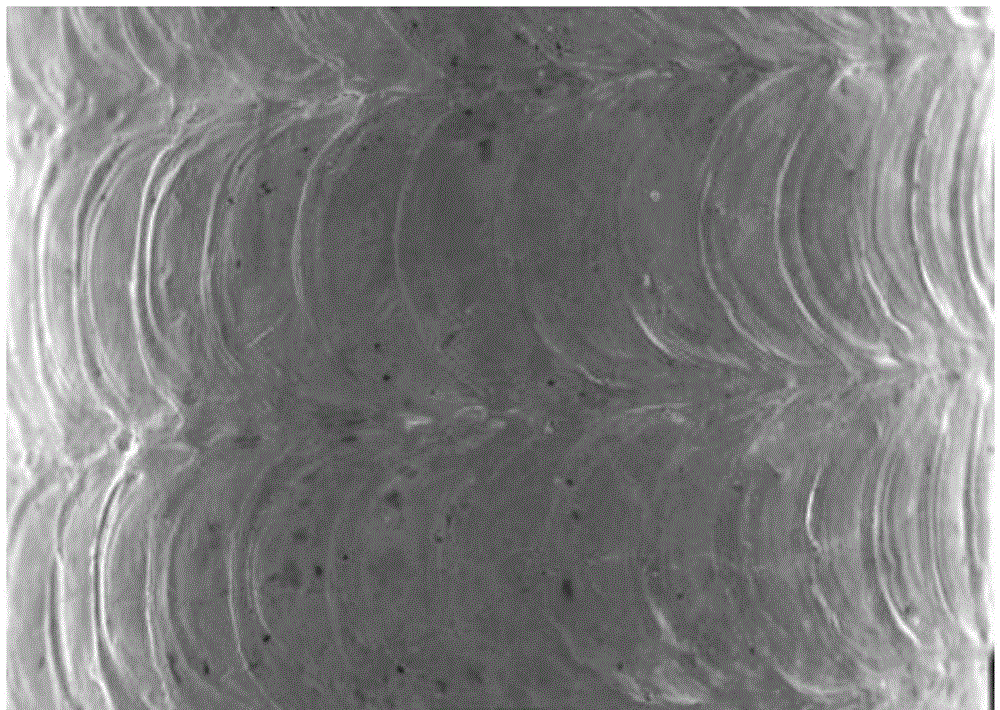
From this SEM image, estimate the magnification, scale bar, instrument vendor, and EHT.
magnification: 0.03 K X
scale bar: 500000 nm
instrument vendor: JEOL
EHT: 20 kV